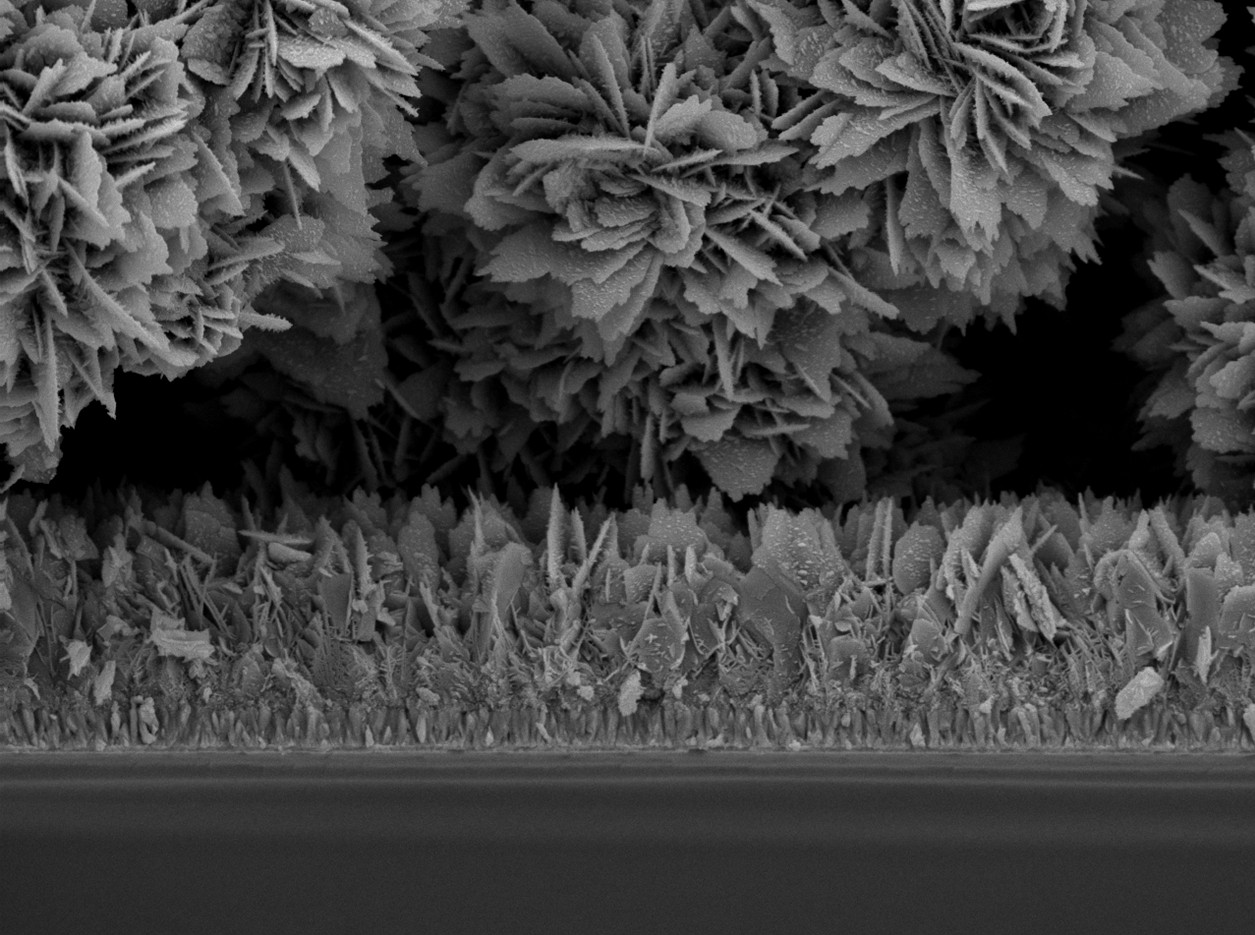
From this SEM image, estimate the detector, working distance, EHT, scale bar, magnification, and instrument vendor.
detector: SEI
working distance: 9.6 mm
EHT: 5 kV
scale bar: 1000 nm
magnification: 10 K X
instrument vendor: JEOL